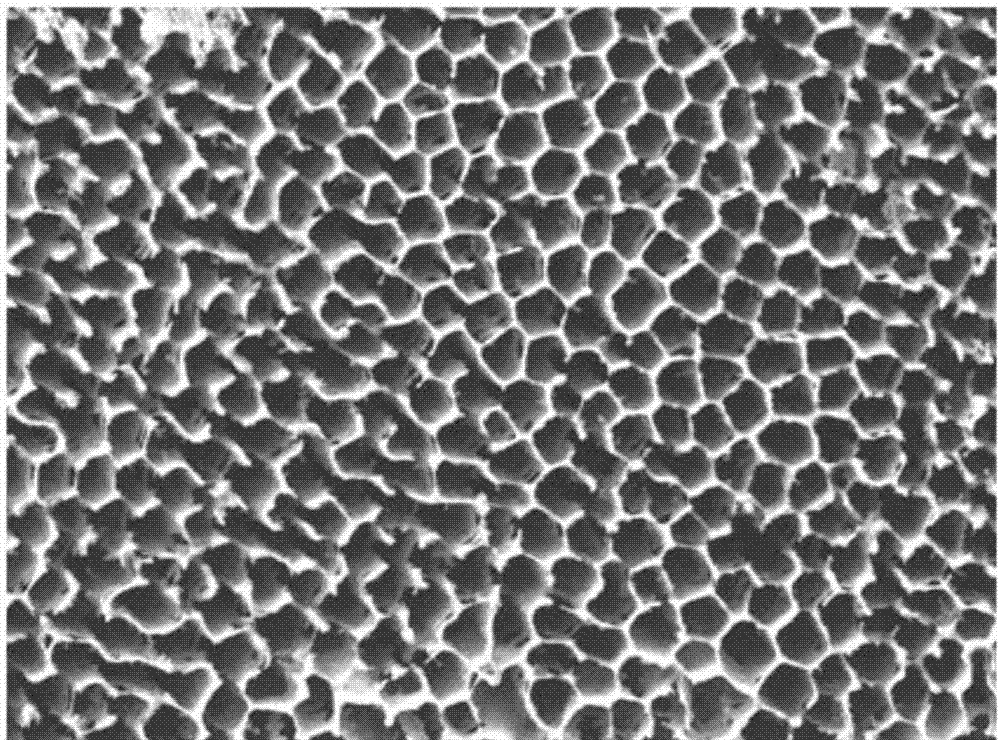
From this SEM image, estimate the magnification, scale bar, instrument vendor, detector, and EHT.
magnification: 0.5 K X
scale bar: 10000 nm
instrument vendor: JEOL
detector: LEI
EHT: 15 kV